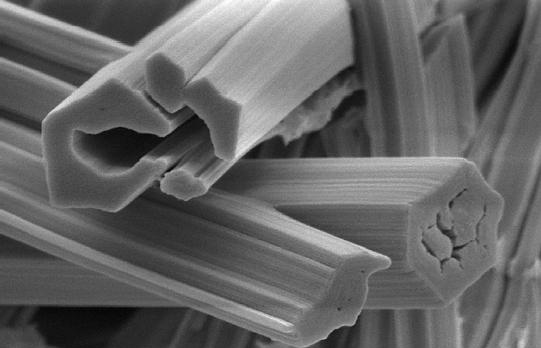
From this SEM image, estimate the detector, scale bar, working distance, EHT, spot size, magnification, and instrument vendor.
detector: SE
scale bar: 2000 nm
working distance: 4.7 mm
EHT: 5 kV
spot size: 2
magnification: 12 K X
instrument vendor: FEI